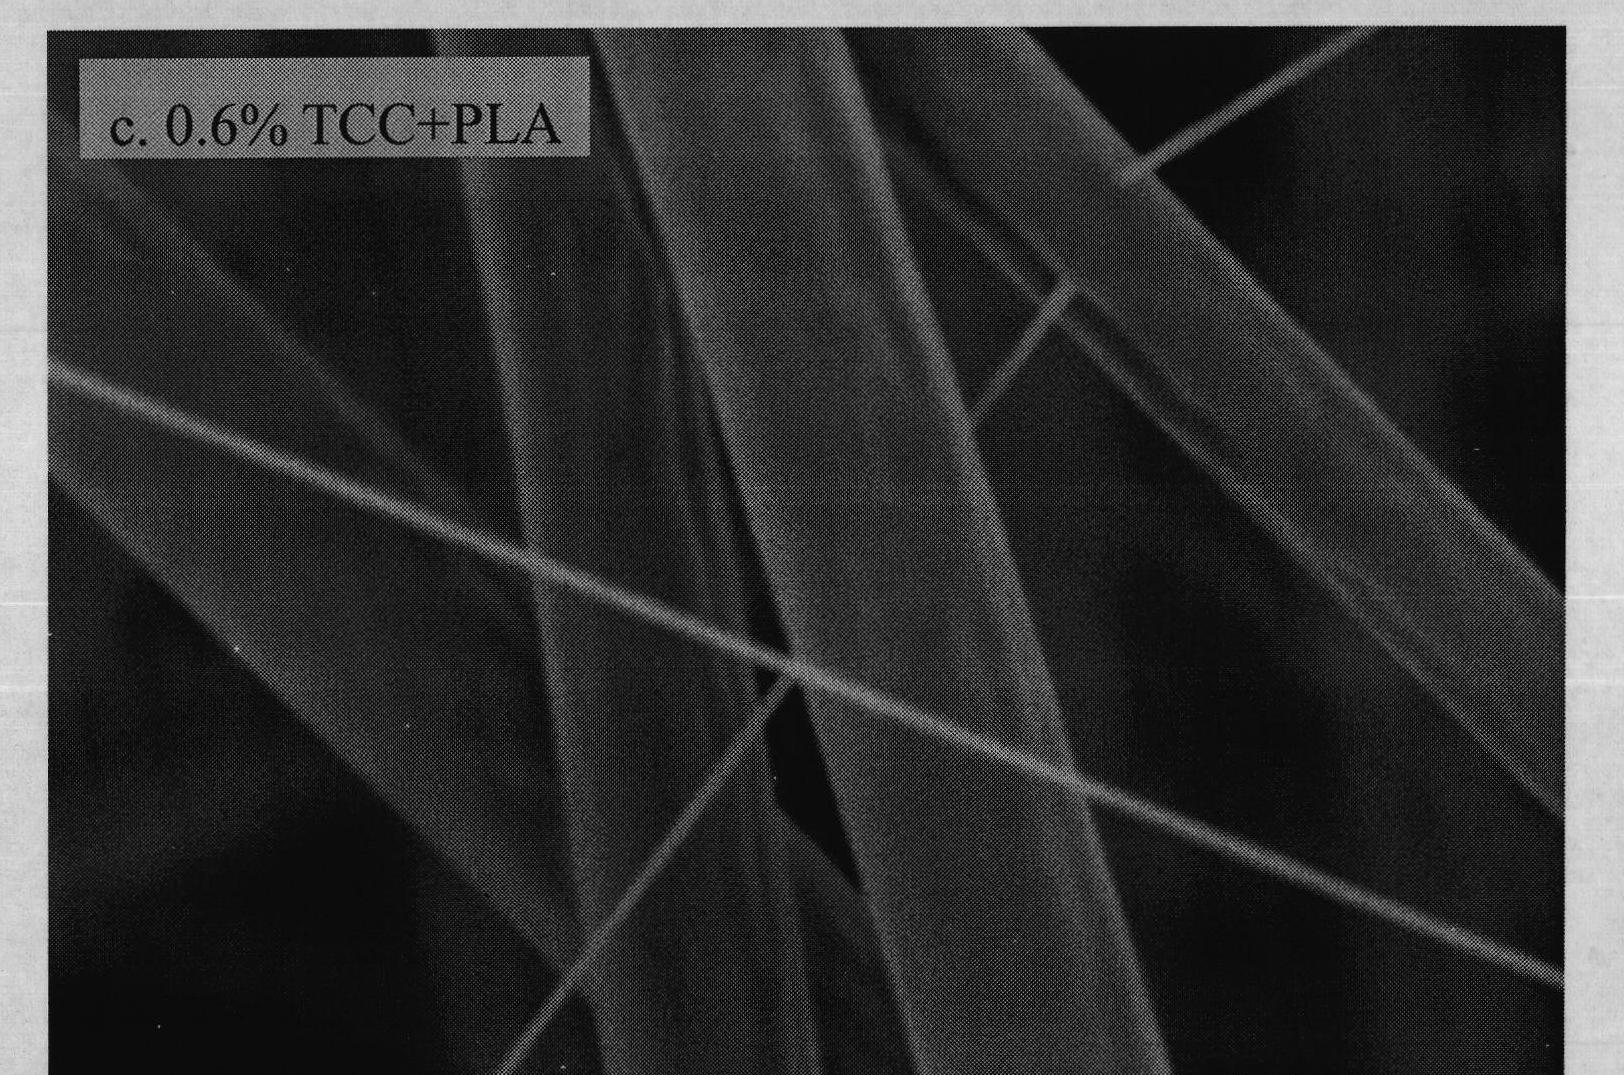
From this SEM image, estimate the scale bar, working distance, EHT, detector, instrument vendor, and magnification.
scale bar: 10000 nm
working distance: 18.2 mm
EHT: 5 kV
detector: SE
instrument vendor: Hitachi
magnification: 5 K X